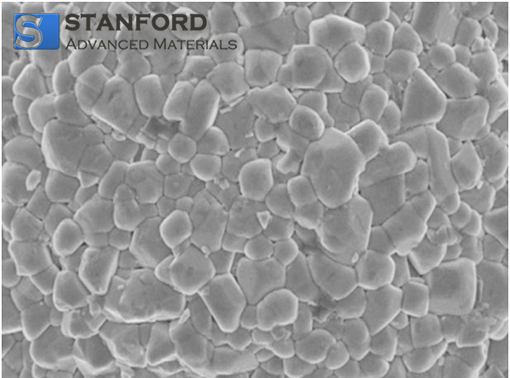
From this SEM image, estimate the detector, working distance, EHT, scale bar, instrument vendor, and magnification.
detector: SE(M)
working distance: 8.1 mm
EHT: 3 kV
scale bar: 2000 nm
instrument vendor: Hitachi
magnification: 20 K X